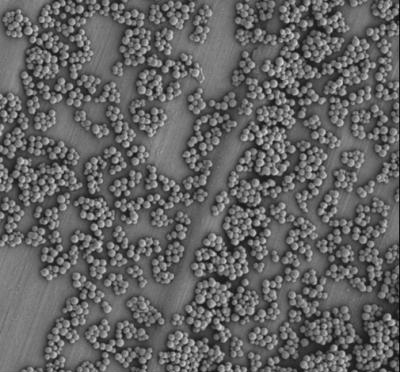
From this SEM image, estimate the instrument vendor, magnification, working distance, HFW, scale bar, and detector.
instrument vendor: FEI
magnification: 10 K X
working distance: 6.9 mm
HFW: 41.4 µm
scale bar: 10000 nm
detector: ETD